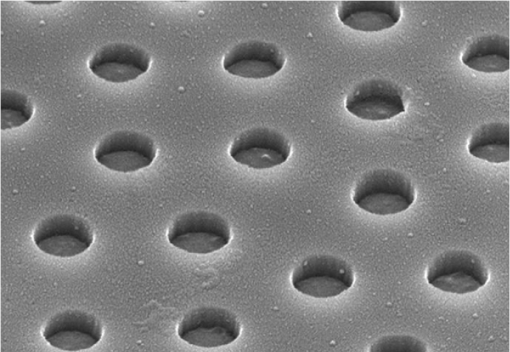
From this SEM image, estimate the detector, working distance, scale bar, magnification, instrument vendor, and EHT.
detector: SE(U)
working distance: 11.6 mm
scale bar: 1000 nm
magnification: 45 K X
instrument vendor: Hitachi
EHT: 10 kV